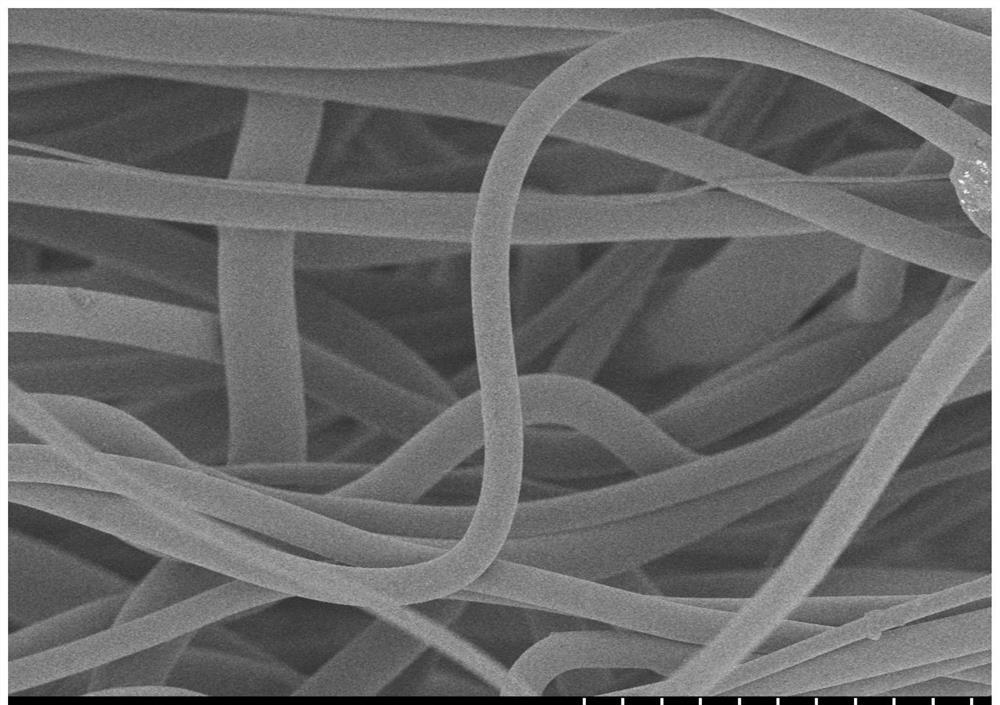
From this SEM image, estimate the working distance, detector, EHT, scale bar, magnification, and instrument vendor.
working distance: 7.5 mm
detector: SE(M)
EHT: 3 kV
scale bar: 50000 nm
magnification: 1 K X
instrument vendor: Hitachi